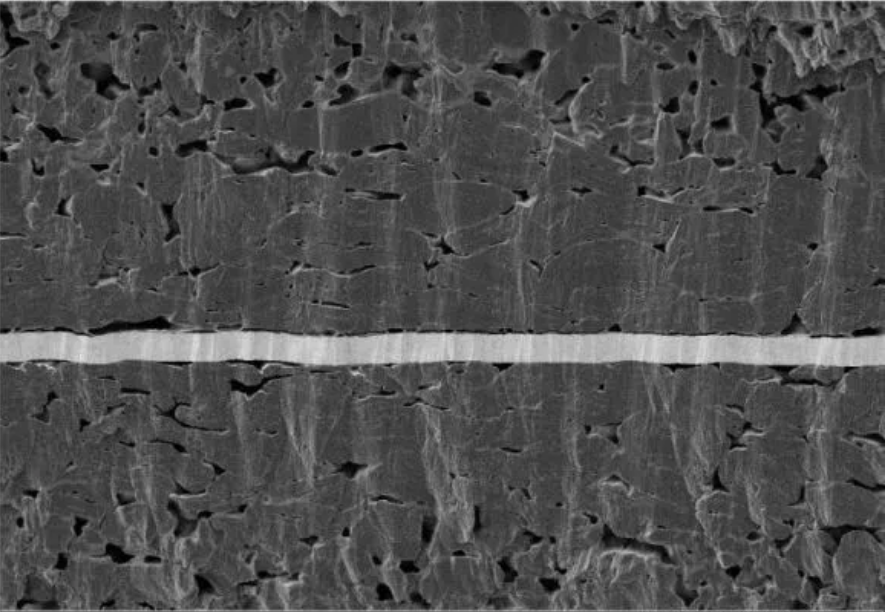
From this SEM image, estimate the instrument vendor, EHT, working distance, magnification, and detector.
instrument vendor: Zeiss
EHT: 3 kV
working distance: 4.1 mm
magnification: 0.601 K X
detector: SE2